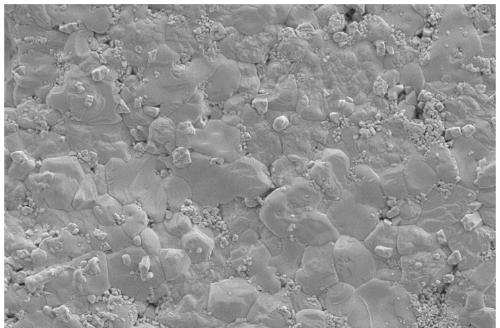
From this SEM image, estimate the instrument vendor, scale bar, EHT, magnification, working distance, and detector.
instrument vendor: Zeiss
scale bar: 2000 nm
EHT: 5 kV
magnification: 5 K X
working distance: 8.2 mm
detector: SE2